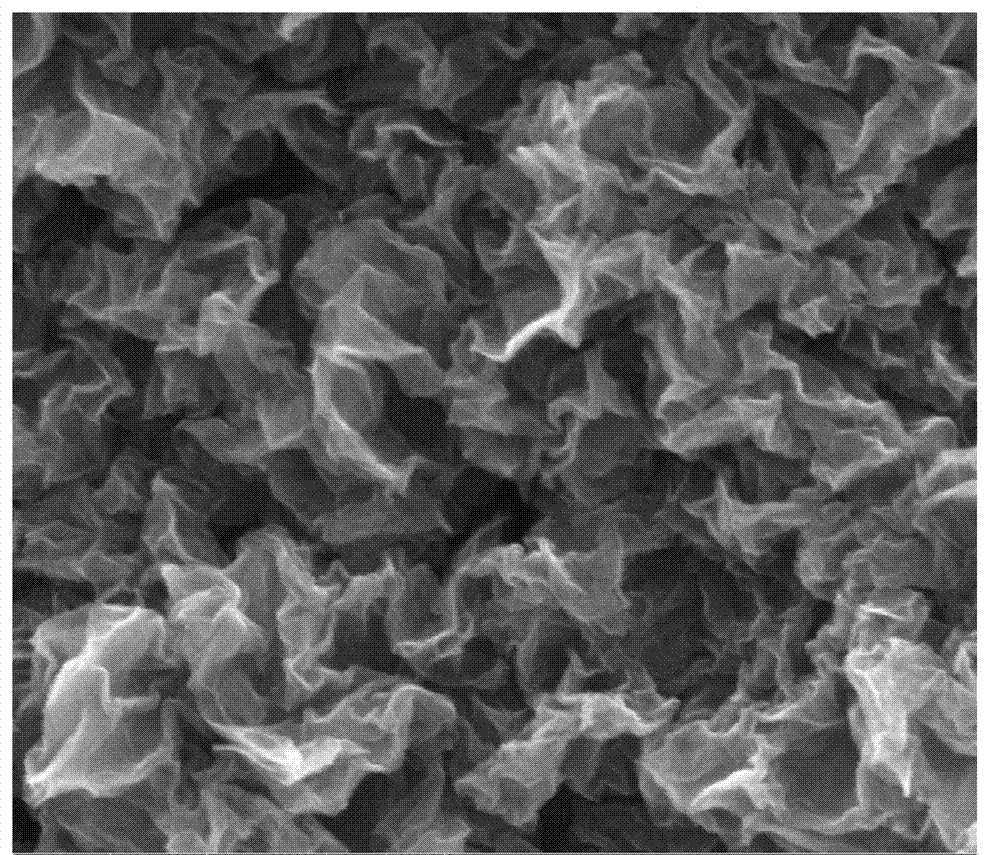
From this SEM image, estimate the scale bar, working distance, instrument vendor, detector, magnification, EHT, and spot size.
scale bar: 1000 nm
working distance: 9 mm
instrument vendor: FEI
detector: ETD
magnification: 100 K X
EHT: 5 kV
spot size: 3